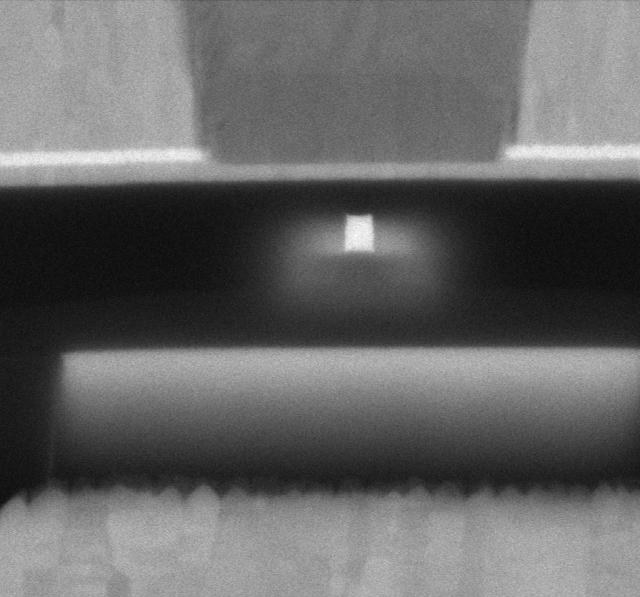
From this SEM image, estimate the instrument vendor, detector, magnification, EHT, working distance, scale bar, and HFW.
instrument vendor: Thermo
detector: MD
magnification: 568.609 K X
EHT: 5 kV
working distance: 2 mm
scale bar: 100 nm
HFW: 0.635 µm